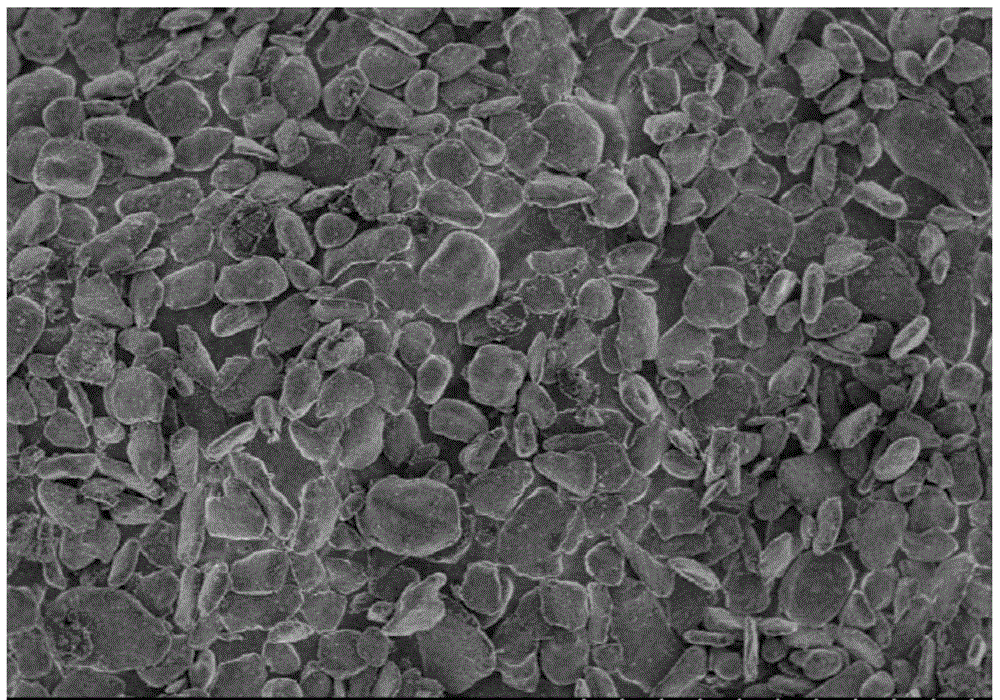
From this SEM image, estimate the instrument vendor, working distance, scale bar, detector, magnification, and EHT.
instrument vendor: Hitachi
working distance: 7.7 mm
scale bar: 100000 nm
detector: SE(M)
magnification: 0.5 K X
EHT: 3 kV